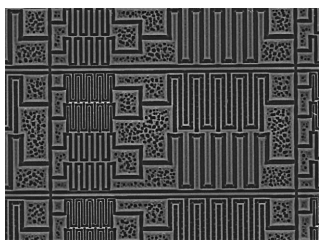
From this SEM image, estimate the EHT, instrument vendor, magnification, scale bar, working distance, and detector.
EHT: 15 kV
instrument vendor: JEOL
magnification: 0.5 K X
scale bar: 10000 nm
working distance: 10.2 mm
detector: TOPO